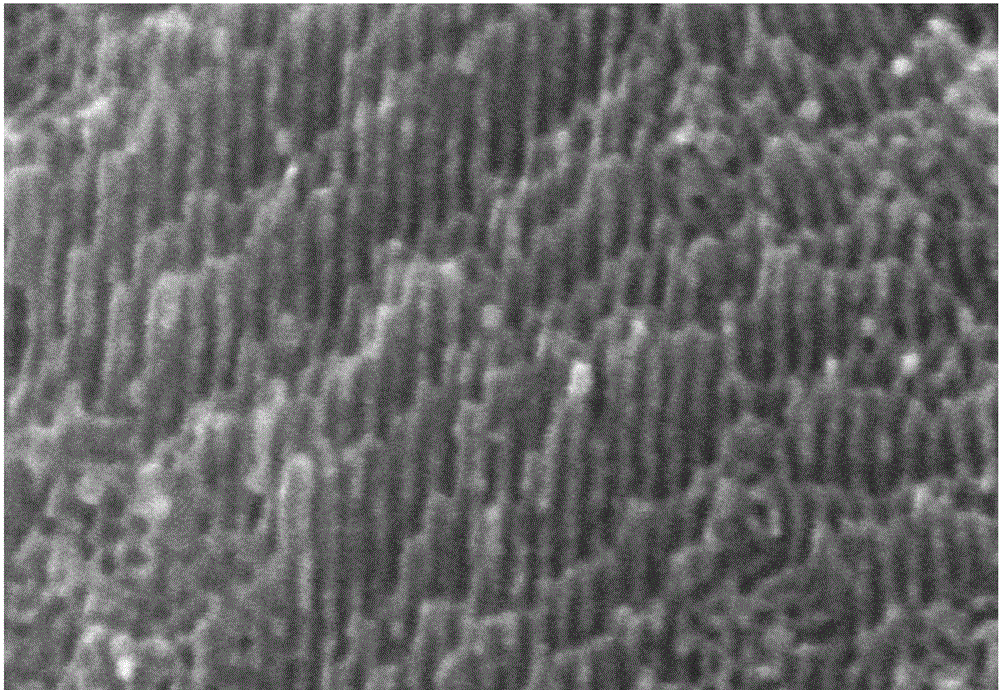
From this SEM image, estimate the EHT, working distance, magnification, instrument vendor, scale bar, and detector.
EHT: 5 kV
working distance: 8.1 mm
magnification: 300 K X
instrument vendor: Hitachi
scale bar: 100 nm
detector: SE(U)